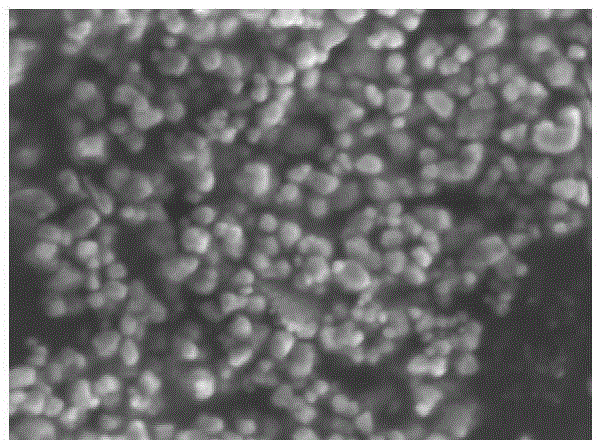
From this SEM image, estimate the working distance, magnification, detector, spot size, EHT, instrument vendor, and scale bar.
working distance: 5.1 mm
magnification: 25.719 K X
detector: TLD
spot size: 4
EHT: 5 kV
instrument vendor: FEI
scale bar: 1000 nm